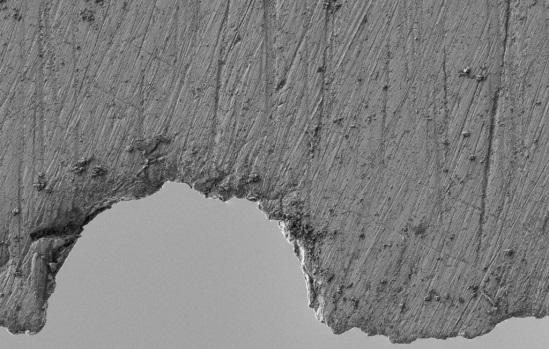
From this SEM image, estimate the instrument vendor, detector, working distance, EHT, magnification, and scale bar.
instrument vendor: Zeiss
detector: SE2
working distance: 4.5 mm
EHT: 1 kV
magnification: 1.5 K X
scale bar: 10000 nm